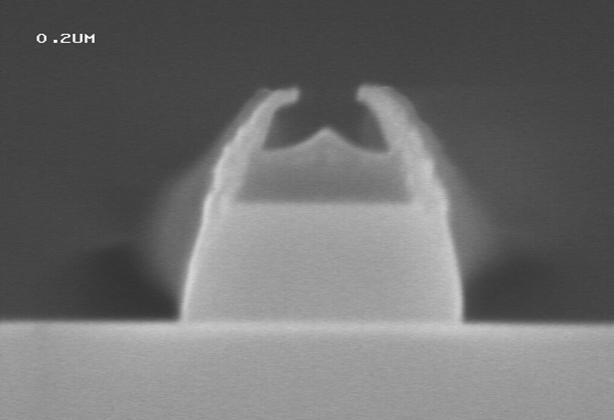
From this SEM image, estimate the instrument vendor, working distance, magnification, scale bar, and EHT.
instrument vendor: JEOL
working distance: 3 mm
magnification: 100 K X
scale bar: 200 nm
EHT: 3 kV